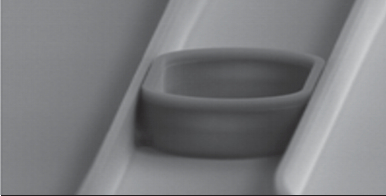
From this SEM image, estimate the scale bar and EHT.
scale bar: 3000 nm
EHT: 10 kV